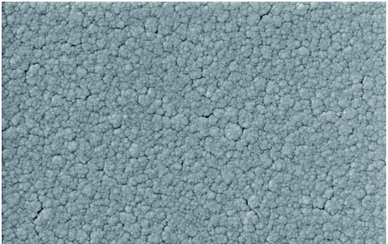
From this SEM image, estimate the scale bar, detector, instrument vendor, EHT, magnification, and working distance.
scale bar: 300 nm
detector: InLens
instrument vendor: Zeiss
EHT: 5 kV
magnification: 50 K X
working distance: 7.1 mm